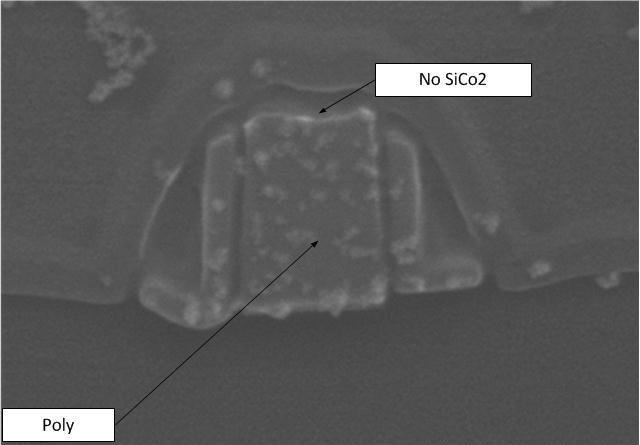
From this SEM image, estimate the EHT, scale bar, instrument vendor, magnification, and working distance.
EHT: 10 kV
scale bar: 200 nm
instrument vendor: Hitachi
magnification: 200 K X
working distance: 8.1 mm